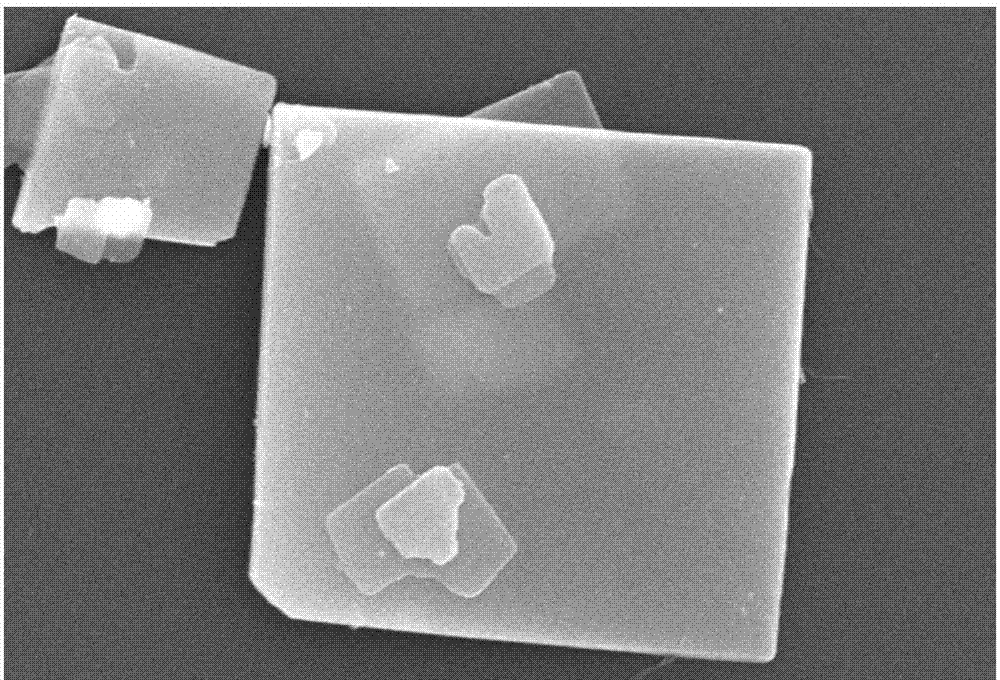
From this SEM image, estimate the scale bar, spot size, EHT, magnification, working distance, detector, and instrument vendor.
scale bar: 1000 nm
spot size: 30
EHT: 15 kV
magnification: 14 K X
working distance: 10 mm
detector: SEI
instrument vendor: JEOL